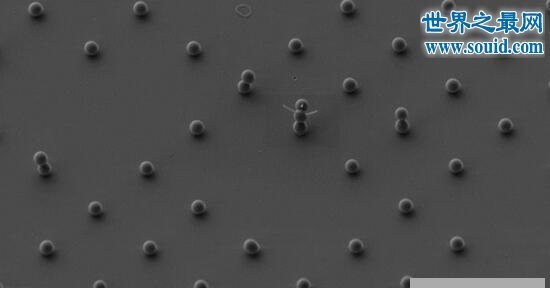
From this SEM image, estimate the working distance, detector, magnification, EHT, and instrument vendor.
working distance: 4.4 mm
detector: SE2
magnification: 3 K X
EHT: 3 kV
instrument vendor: Zeiss